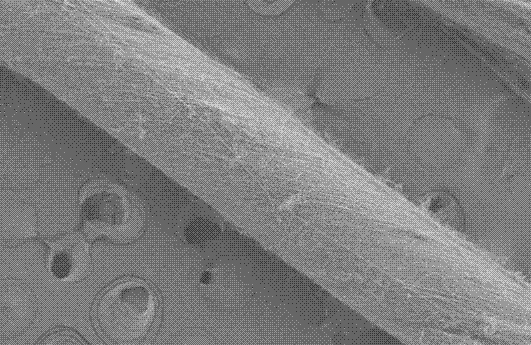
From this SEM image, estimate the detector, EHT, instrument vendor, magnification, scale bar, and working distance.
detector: SE2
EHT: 5 kV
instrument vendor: Zeiss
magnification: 0.15 K X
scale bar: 200000 nm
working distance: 8.5 mm